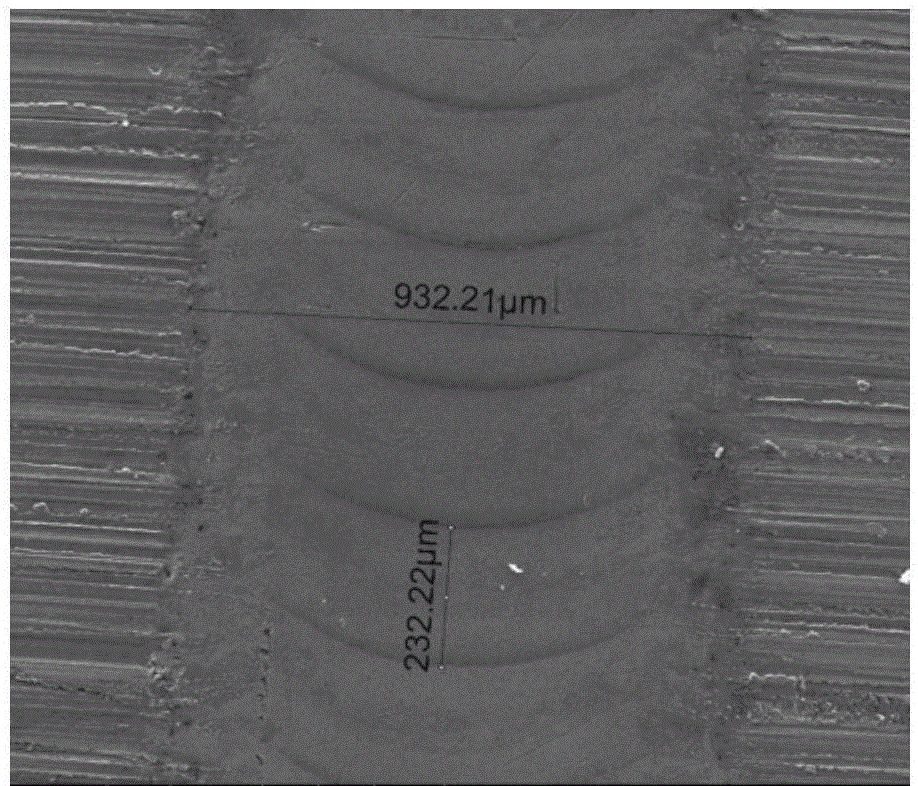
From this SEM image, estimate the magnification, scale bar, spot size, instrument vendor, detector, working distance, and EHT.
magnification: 0.2 K X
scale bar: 500000 nm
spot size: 6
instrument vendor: FEI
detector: ETD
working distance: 9.4 mm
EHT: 20 kV